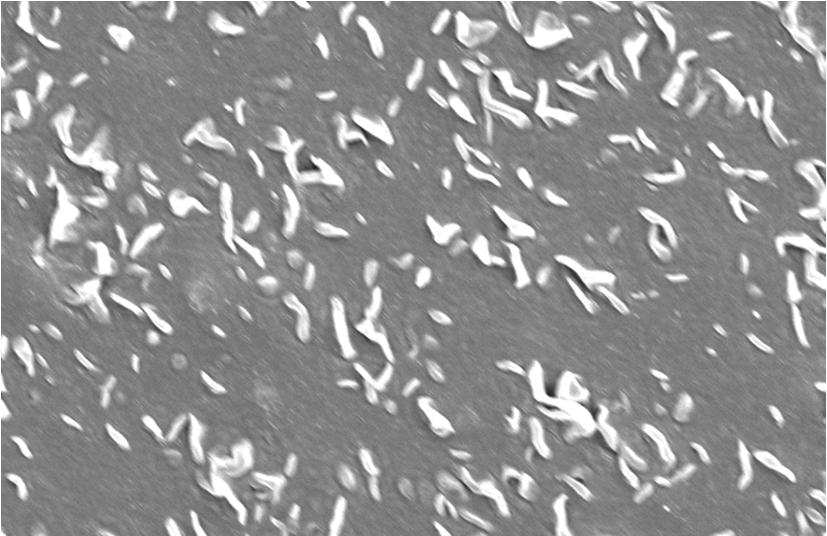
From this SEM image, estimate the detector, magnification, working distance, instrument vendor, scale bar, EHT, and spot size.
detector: SE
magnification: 2 K X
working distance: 22.4 mm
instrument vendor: FEI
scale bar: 10000 nm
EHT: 15 kV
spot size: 3